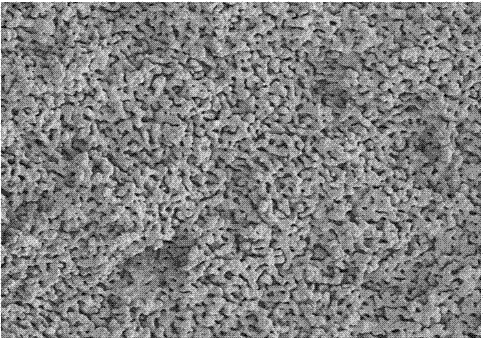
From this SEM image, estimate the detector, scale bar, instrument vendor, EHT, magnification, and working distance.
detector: SE(M,LA0)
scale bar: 20000 nm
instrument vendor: Hitachi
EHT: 5 kV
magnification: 2 K X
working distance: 8.4 mm